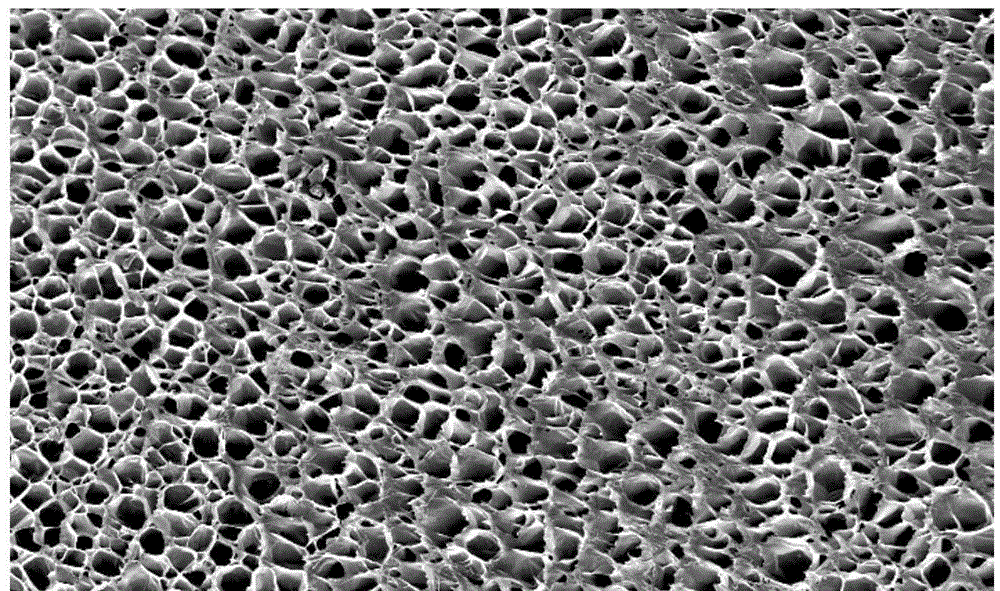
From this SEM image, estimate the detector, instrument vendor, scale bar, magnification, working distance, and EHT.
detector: SE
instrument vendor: FEI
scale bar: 200000 nm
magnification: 0.1 K X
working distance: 30 mm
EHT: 10 kV